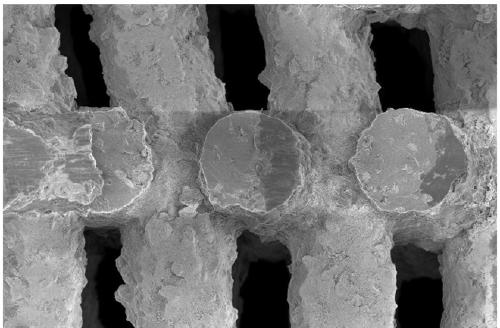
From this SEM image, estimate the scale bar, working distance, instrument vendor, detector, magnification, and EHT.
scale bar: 100000 nm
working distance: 5 mm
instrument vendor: Zeiss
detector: InLens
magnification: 0.15 K X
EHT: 5 kV